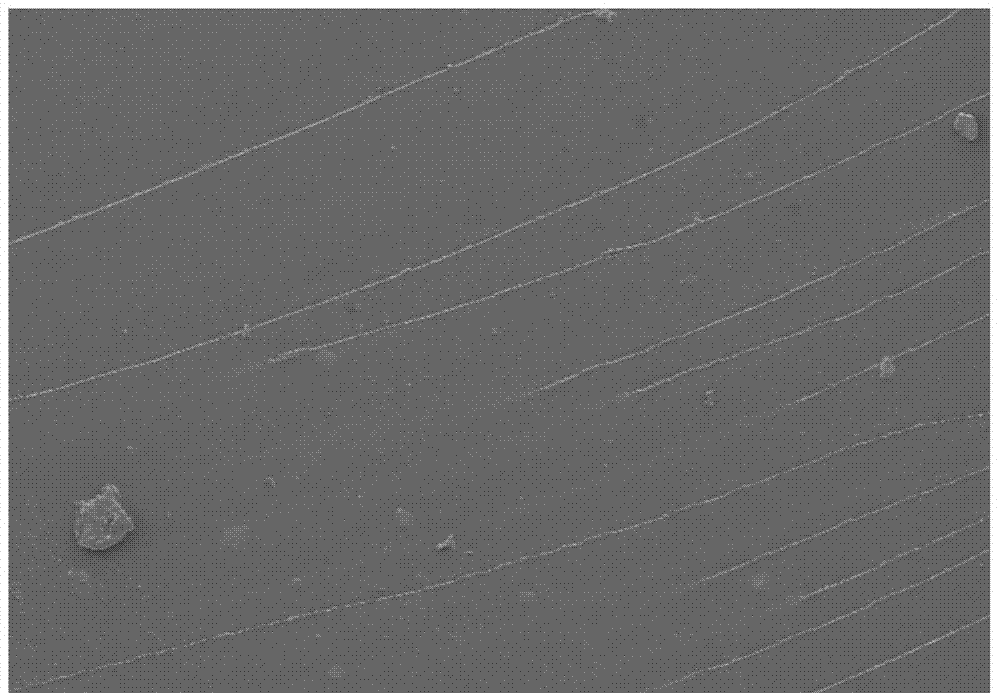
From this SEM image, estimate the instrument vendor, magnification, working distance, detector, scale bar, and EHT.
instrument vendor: Hitachi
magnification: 0.1 K X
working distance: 11.2 mm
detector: SE(M)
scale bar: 500000 nm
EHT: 5 kV